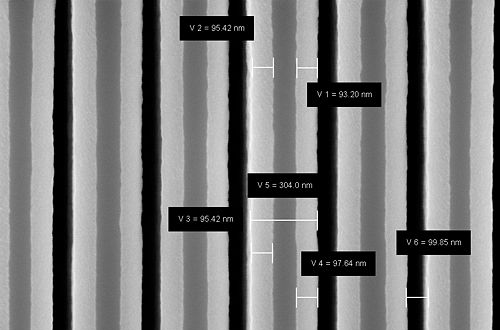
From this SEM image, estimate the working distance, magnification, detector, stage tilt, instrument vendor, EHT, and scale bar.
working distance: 3.4 mm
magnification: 132.47 K X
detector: InLens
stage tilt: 0°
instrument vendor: Zeiss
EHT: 5 kV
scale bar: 200 nm